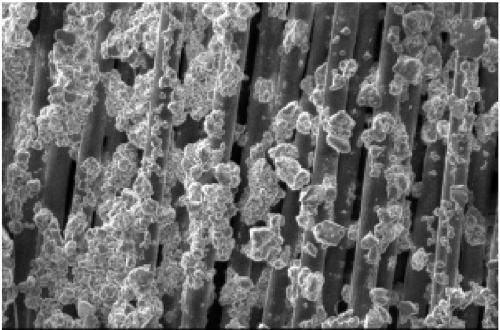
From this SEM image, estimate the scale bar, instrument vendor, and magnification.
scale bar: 50000 nm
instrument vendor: JEOL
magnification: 0.5 K X